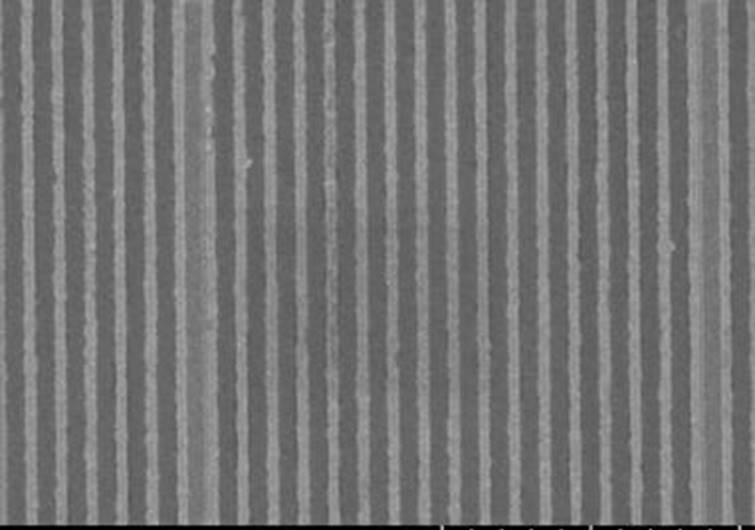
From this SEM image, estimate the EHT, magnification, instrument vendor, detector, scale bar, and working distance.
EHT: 1 kV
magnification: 10 K X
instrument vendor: Hitachi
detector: SE(U)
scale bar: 5000 nm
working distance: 8.4 mm